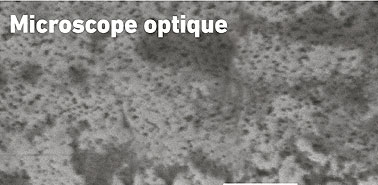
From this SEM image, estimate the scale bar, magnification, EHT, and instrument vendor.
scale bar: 5000 nm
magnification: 3 K X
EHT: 15 kV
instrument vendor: JEOL